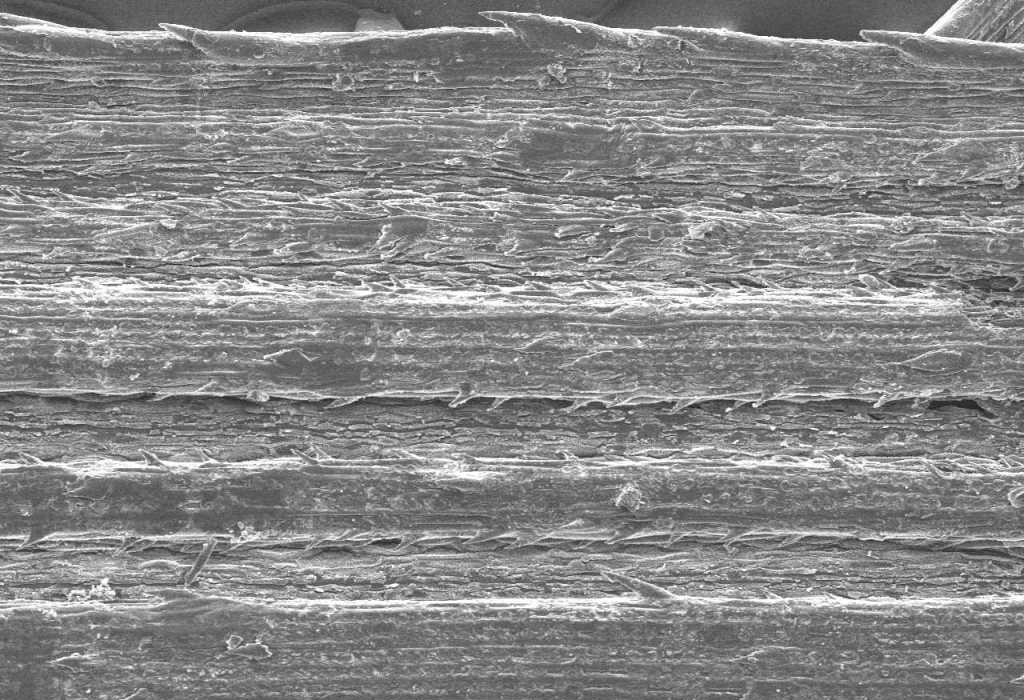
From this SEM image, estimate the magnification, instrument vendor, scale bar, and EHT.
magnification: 0.14 K X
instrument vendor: JEOL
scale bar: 100000 nm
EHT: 9 kV